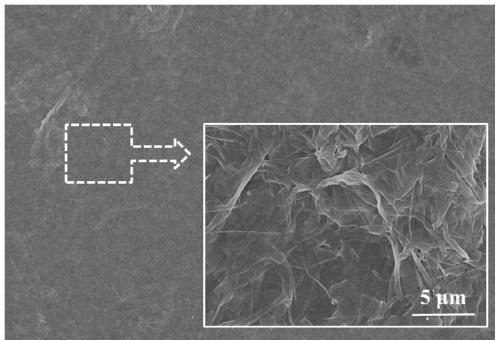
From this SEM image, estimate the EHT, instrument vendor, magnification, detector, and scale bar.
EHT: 8 kV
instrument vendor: Hitachi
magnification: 2 K X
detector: SE(U)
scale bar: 20000 nm